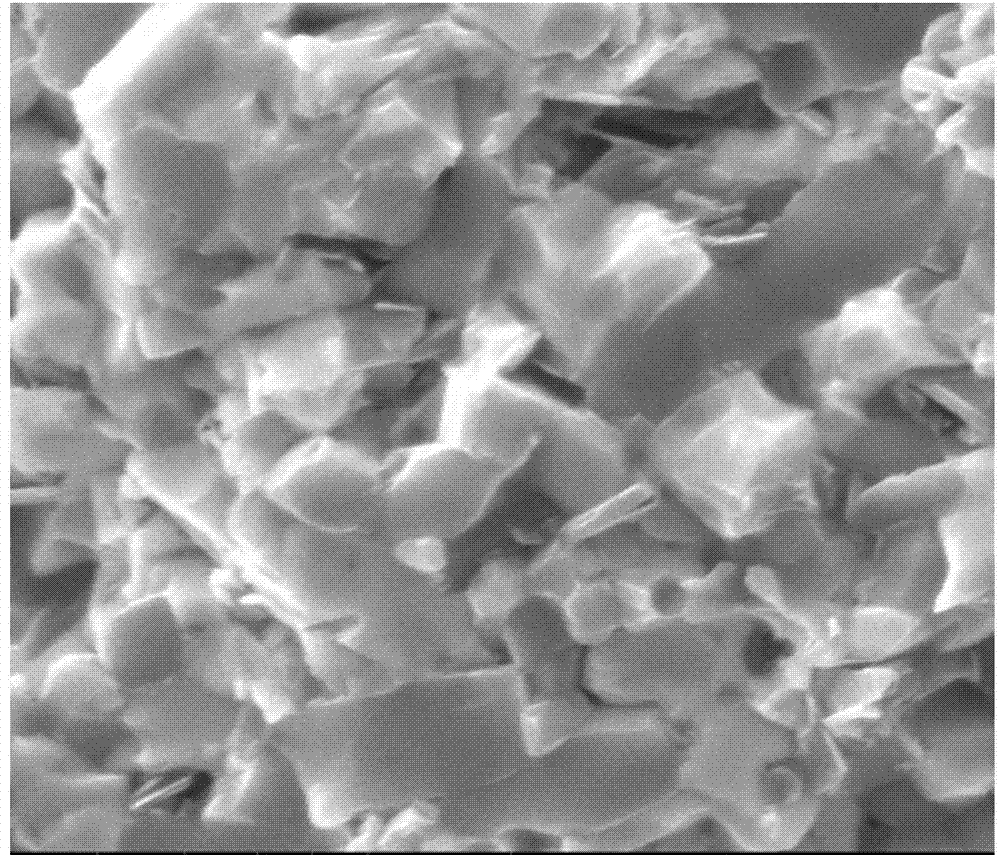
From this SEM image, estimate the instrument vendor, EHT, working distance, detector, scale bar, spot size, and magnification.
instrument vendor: FEI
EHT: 20 kV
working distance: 9.2 mm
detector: ETD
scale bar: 10000 nm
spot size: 3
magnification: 6 K X